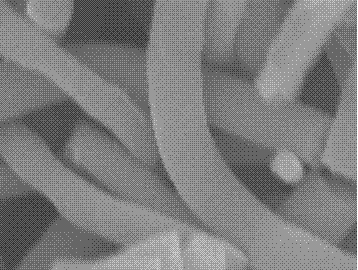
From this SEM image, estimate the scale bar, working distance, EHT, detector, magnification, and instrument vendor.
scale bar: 100 nm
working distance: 4.5 mm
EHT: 6 kV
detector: SEI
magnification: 100 K X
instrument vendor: JEOL